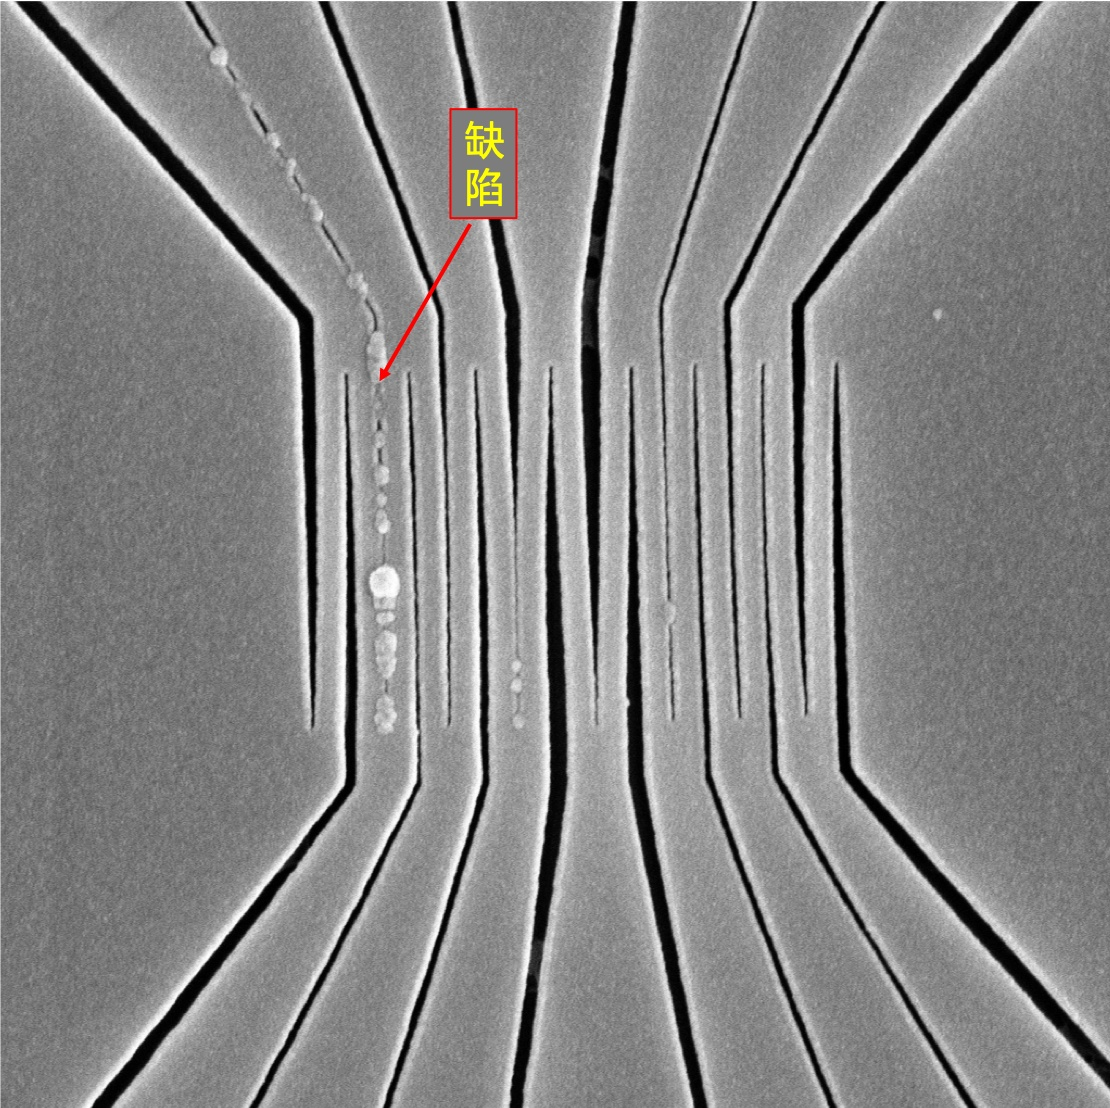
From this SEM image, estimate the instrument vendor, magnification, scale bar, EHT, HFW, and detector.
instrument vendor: other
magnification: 50 K X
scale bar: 1000 nm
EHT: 10 kV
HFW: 5.37 µm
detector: SED